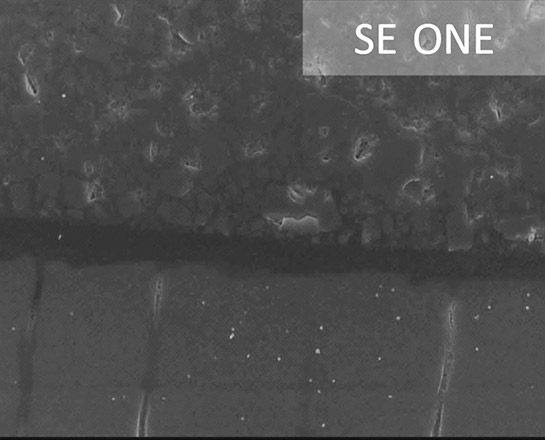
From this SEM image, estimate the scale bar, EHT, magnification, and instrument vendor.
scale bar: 10000 nm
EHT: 15 kV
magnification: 1.5 K X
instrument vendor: JEOL